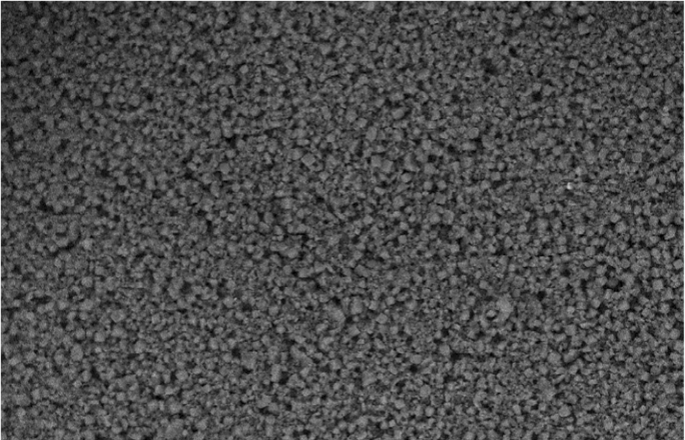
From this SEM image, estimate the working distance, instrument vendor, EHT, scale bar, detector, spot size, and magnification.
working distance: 9.1 mm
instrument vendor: FEI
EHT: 15 kV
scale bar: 50000 nm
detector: BSE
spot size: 5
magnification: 0.65 K X